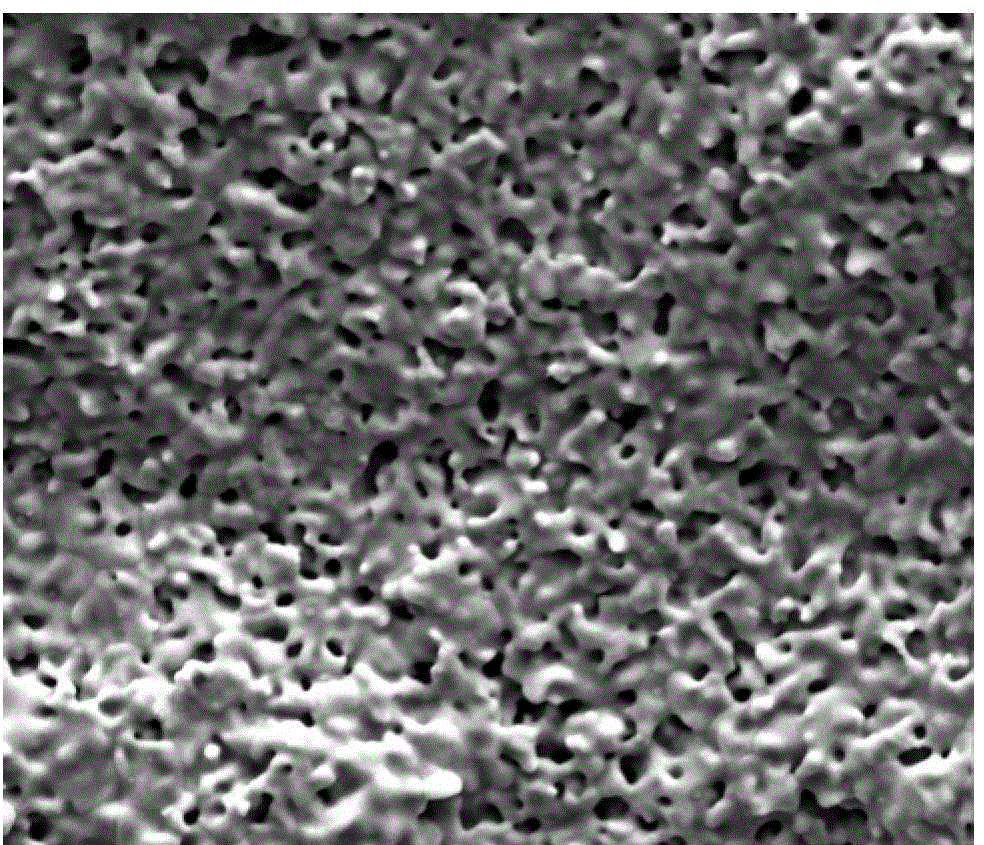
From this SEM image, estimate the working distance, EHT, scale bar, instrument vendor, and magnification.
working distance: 13.6 mm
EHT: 20 kV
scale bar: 50000 nm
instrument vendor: FEI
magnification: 1.3 K X